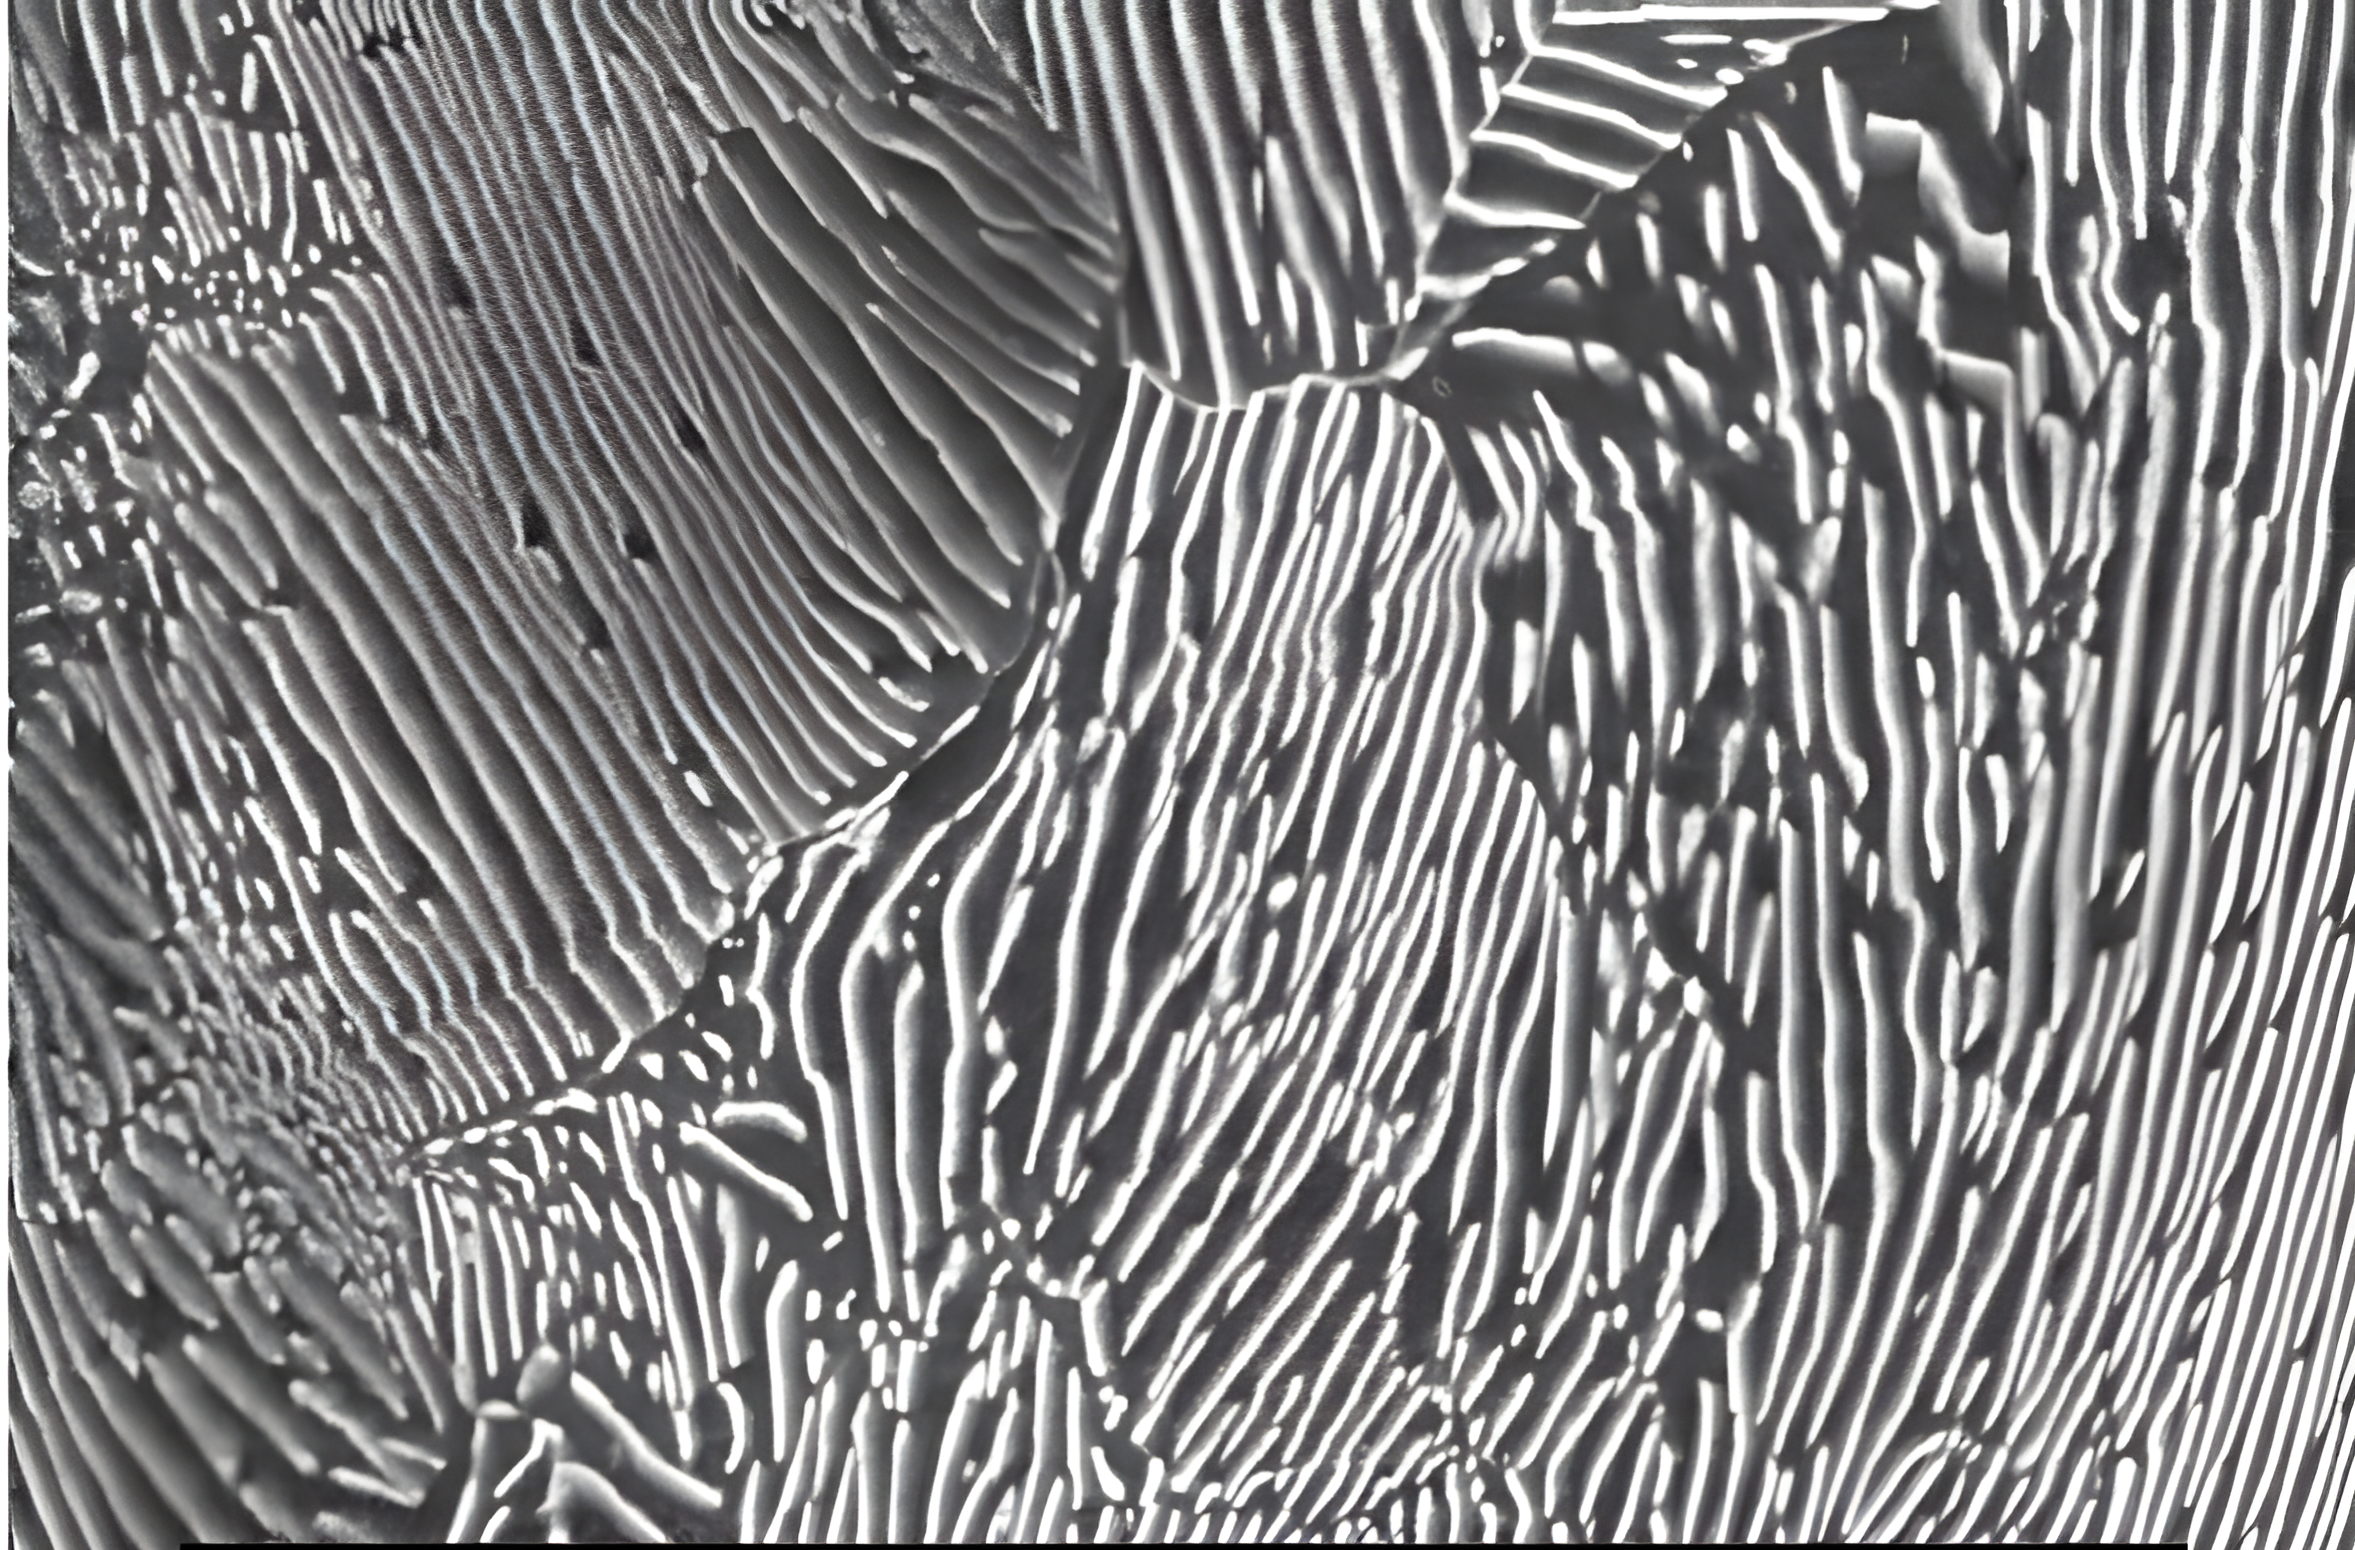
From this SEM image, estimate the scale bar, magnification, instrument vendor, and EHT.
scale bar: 5000 nm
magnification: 5 K X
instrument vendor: JEOL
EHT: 20 kV